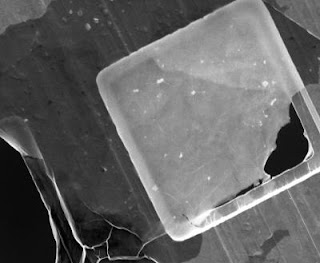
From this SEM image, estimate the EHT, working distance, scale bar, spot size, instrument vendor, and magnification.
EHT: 5 kV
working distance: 10.7 mm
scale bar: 40000 nm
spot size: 3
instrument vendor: FEI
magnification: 2 K X